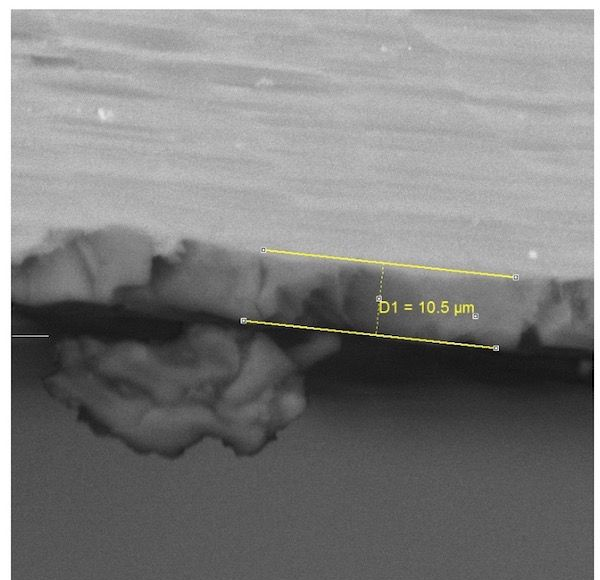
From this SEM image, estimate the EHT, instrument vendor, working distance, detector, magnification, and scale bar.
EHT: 25 kV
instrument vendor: Tescan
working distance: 25.94 mm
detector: BSE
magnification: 2.34 K X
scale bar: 20000 nm